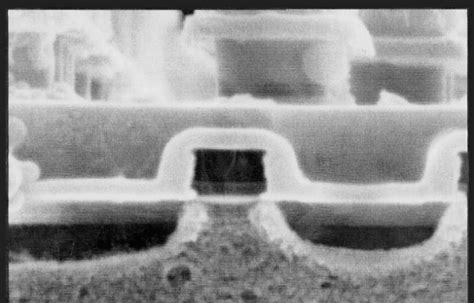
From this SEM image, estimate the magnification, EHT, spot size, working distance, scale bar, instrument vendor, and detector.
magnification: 40 K X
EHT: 10 kV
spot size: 3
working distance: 10.8 mm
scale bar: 500 nm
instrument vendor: FEI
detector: SE